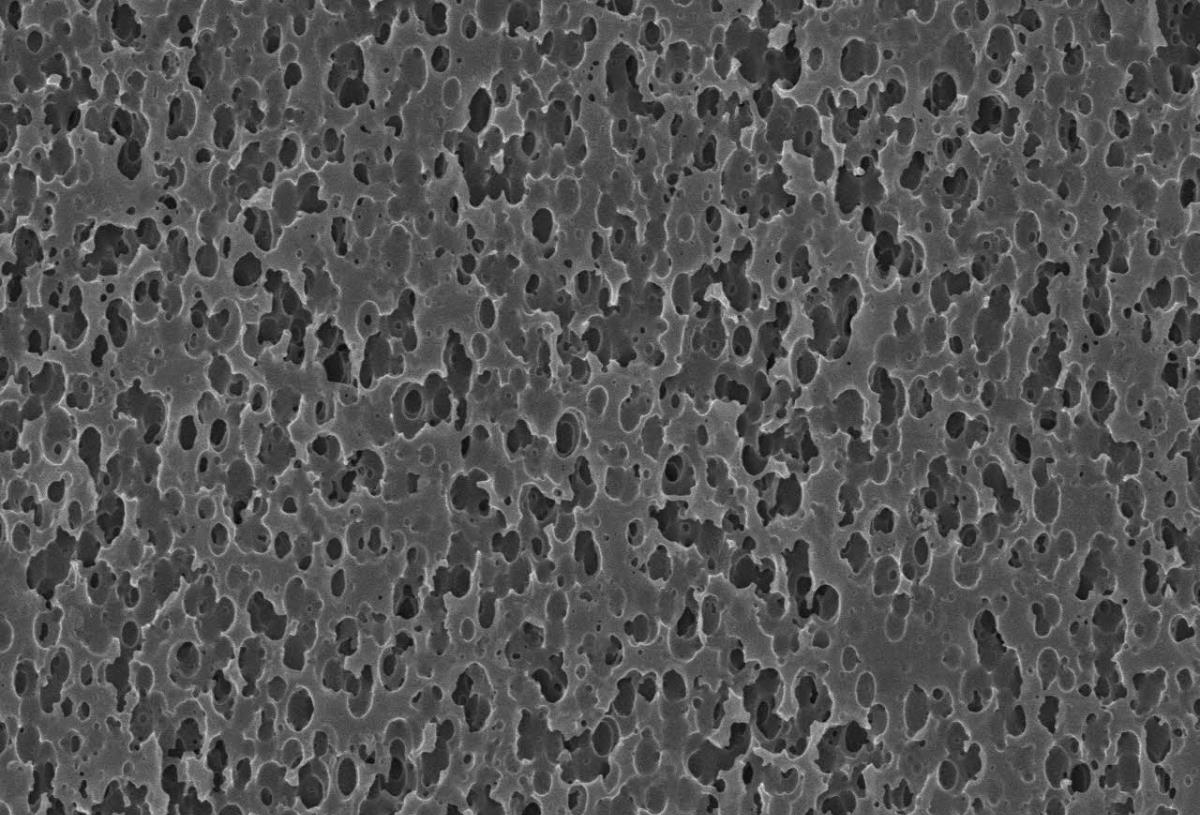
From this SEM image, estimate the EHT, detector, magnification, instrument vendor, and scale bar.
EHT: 15 kV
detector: SEI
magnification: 5 K X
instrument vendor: JEOL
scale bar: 5000 nm